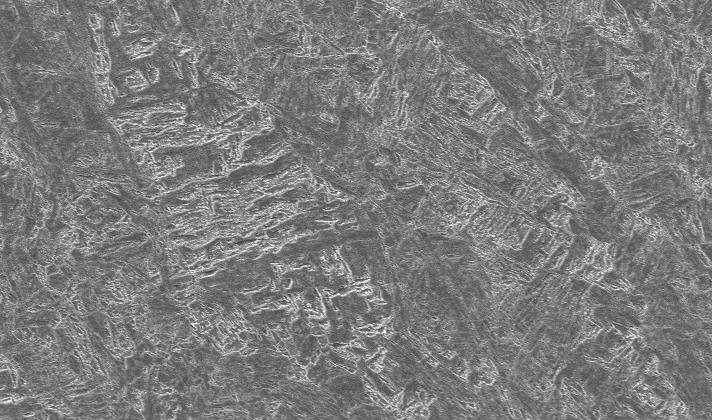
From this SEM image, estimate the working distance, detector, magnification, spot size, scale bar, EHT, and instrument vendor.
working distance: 12.3 mm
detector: SE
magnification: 0.2 K X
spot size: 4.8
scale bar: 100000 nm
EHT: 20 kV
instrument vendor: FEI